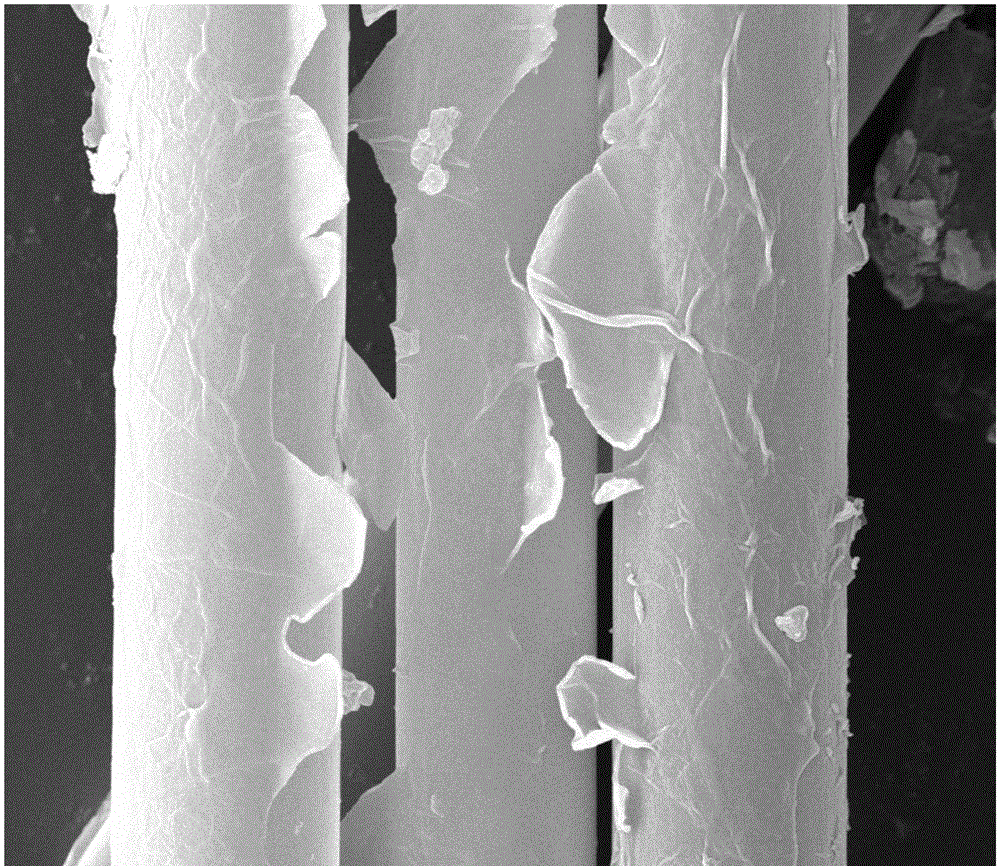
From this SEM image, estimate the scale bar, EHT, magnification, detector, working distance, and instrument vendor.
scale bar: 10000 nm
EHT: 30 kV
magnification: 10 K X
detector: SE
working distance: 10.4 mm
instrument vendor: FEI